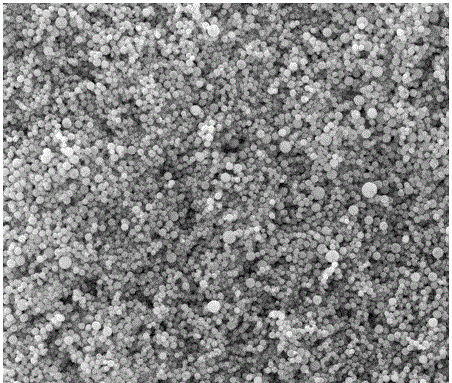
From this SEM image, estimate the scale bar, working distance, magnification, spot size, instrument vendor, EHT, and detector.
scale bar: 20000 nm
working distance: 9.7 mm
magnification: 5 K X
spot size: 3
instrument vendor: FEI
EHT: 30 kV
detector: ETD